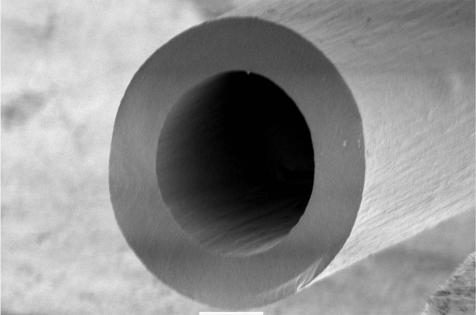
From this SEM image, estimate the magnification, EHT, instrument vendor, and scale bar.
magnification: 0.085 K X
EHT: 5 kV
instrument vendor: JEOL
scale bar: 200000 nm